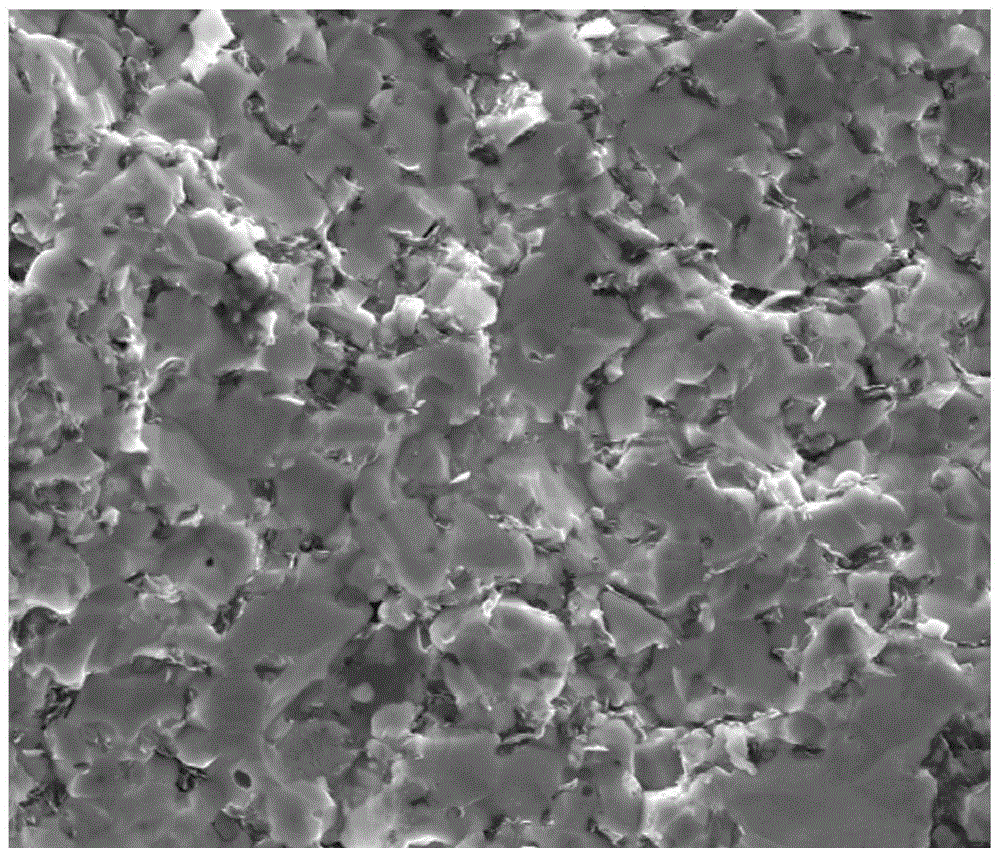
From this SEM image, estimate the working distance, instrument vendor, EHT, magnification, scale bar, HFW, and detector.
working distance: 11 mm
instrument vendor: FEI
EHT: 20 kV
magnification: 5 K X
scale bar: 20000 nm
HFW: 59.7 µm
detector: ETD